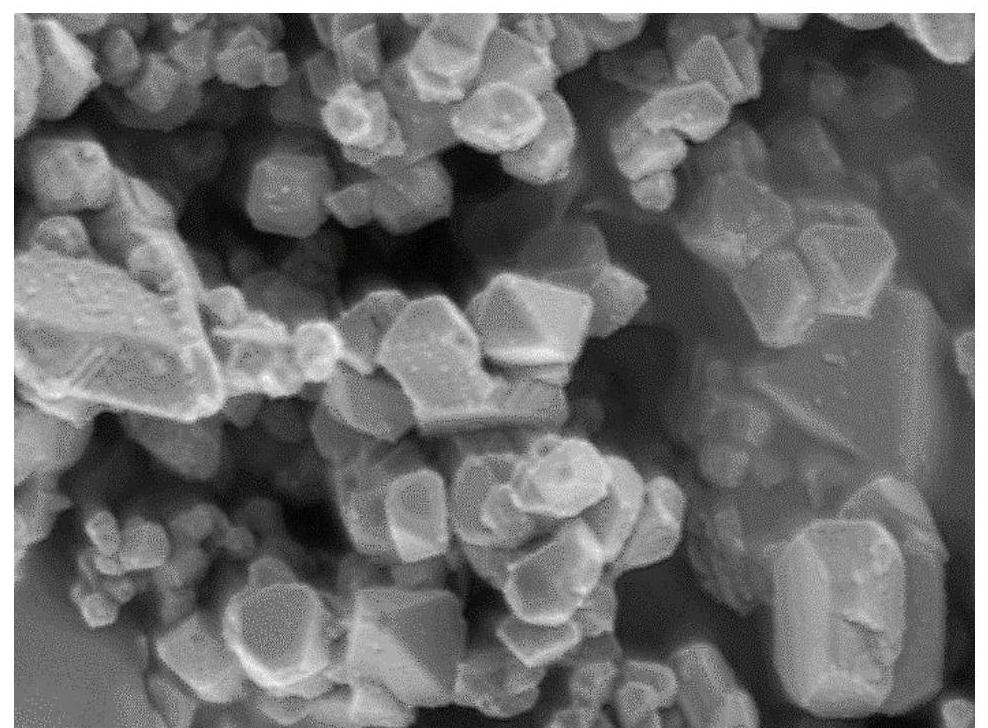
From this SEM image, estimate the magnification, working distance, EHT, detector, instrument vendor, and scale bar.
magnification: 75 K X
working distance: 3.9 mm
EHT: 1 kV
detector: UED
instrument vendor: JEOL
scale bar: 100 nm